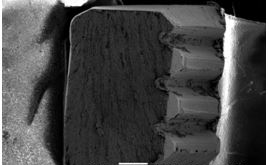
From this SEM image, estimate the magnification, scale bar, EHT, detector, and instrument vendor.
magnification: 0.014 K X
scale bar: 1000000 nm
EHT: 25 kV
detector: SEI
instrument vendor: JEOL